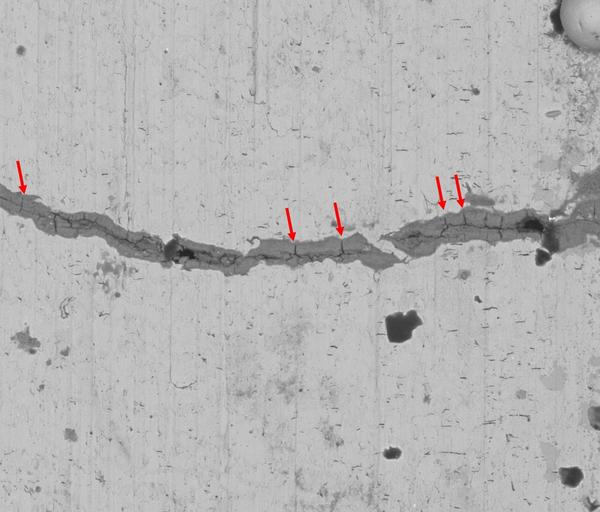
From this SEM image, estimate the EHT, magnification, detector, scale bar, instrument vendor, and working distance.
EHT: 20 kV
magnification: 0.5 K X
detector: BSED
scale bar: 300000 nm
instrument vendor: FEI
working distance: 32.9 mm